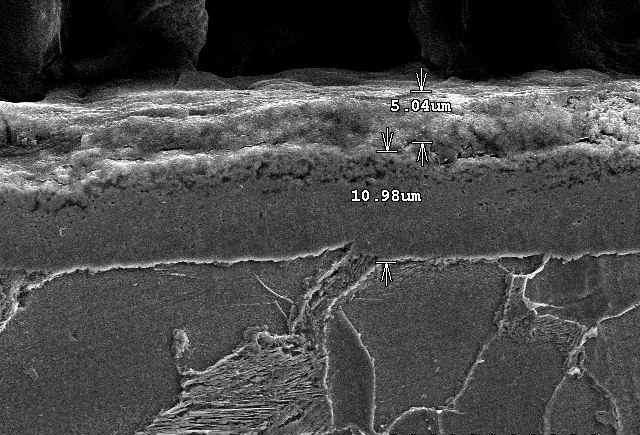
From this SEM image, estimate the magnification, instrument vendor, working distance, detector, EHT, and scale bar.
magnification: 2 K X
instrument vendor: JEOL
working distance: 13.4 mm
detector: SE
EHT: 15 kV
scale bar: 20000 nm